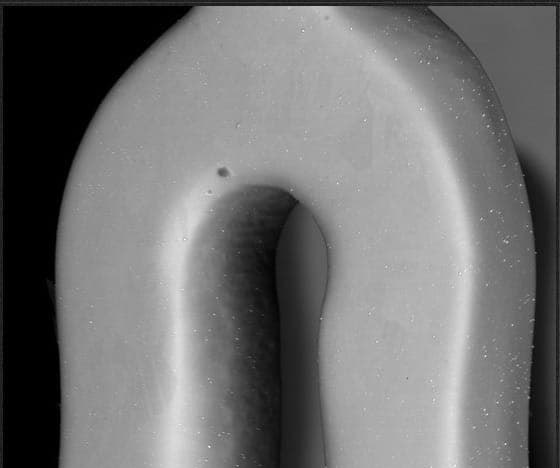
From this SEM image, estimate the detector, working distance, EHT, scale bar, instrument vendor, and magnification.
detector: BSED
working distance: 10 mm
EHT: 25 kV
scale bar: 100000 nm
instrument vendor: FEI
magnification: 1.121 K X